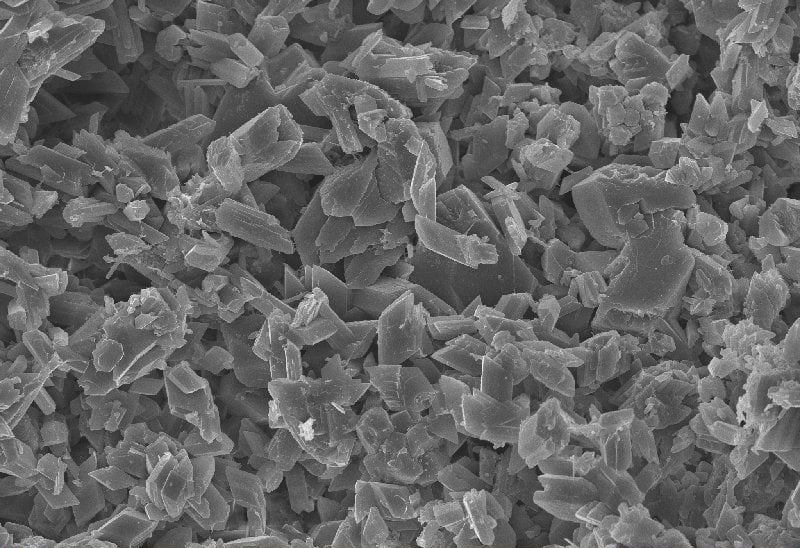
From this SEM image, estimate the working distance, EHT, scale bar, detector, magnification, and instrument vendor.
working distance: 8.4 mm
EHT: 20 kV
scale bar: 10000 nm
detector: InLens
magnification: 1 K X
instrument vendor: Zeiss